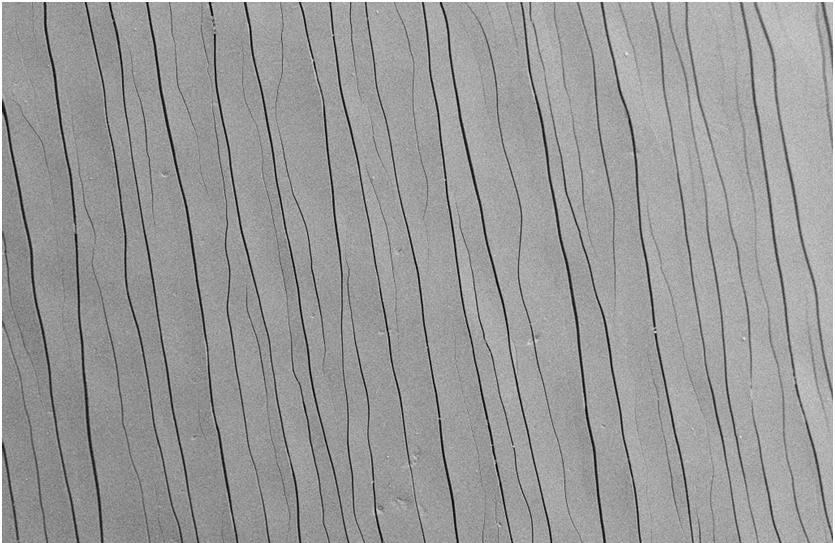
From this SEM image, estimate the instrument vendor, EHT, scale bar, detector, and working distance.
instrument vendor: Zeiss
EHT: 10 kV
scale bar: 100000 nm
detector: SE2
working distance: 5 mm